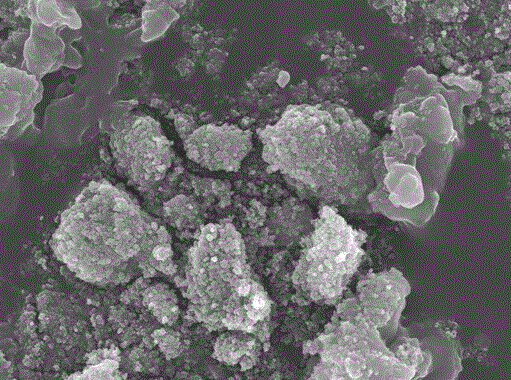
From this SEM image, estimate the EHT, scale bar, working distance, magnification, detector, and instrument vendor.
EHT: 10 kV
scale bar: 1000 nm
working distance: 2.9 mm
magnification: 10 K X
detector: SEI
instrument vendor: JEOL